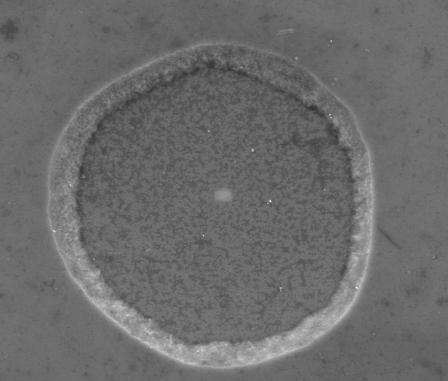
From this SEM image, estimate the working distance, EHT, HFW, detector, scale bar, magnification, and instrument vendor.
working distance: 6.3 mm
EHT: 15 kV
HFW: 85.3 µm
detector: LVSED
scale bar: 20000 nm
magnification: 3.5 K X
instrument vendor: FEI